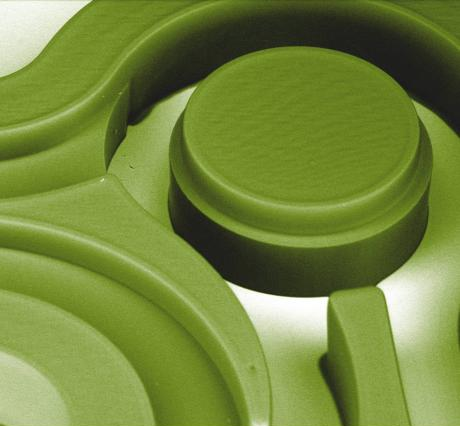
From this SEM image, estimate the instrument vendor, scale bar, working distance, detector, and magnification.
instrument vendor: FEI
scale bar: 500000 nm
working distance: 10 mm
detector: BSED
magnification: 0.202 K X